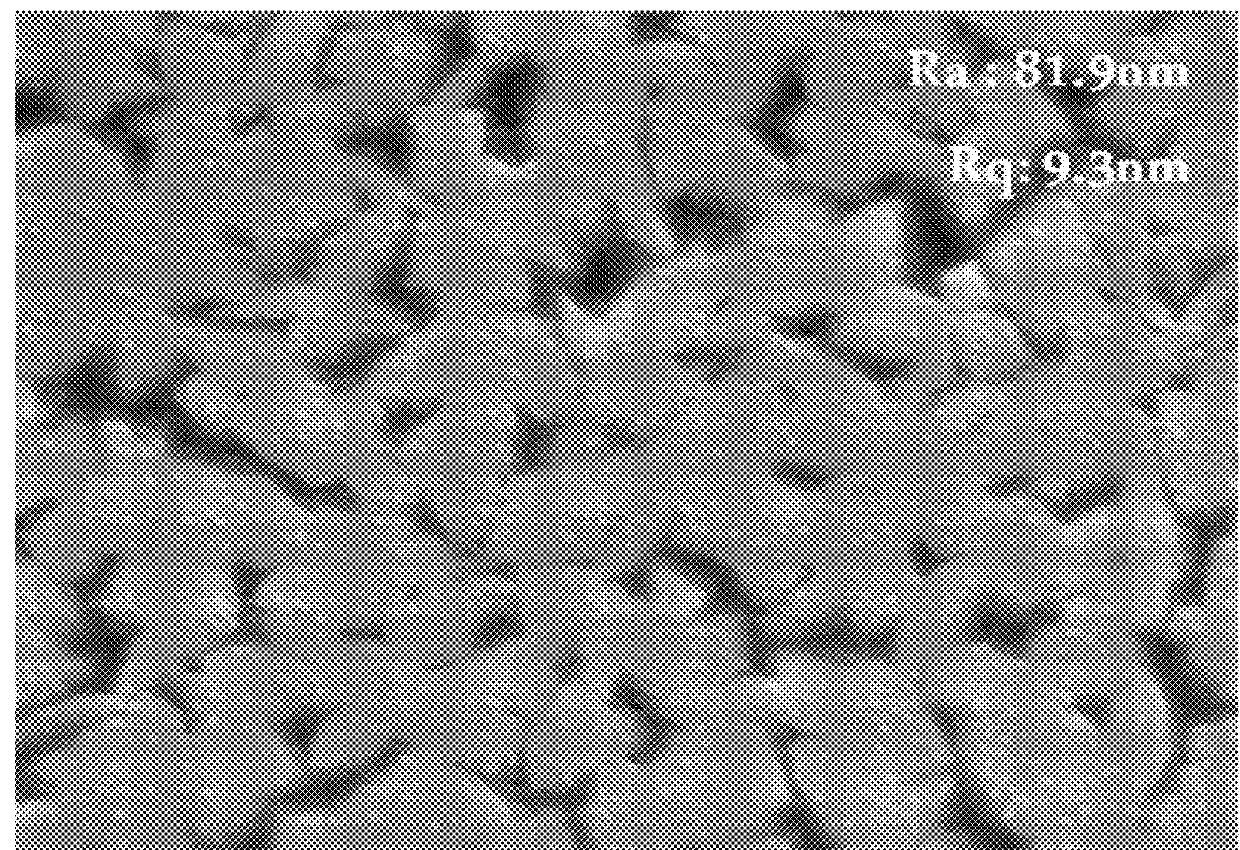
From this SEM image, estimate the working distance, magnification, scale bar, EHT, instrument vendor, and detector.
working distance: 6.8 mm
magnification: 50 K X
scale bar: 100 nm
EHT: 15 kV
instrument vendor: JEOL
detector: SEI